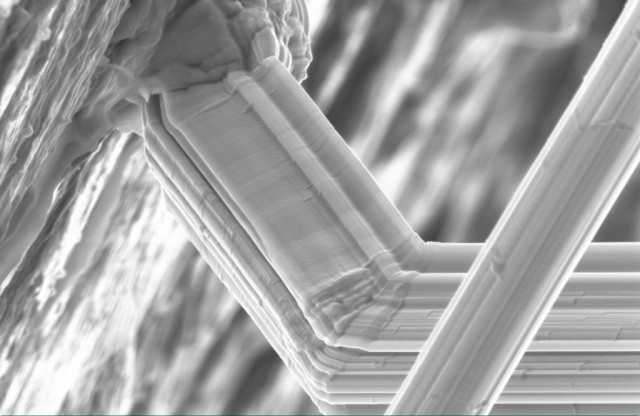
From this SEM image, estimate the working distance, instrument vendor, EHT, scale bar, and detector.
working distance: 34 mm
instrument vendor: Zeiss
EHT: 10 kV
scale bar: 1000 nm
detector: SE2